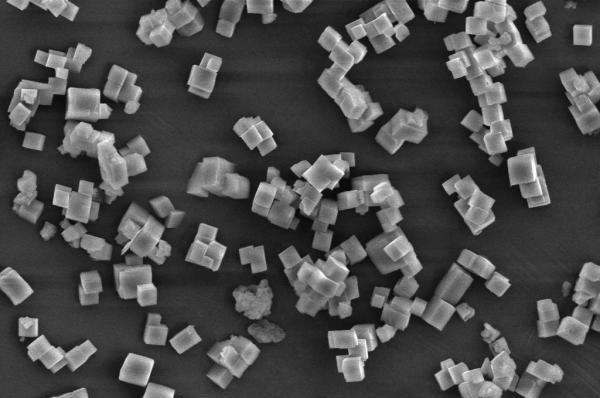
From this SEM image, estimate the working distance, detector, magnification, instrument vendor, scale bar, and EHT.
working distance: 8.5 mm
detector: SE1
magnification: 2 K X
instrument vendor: Zeiss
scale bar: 2000 nm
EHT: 10 kV